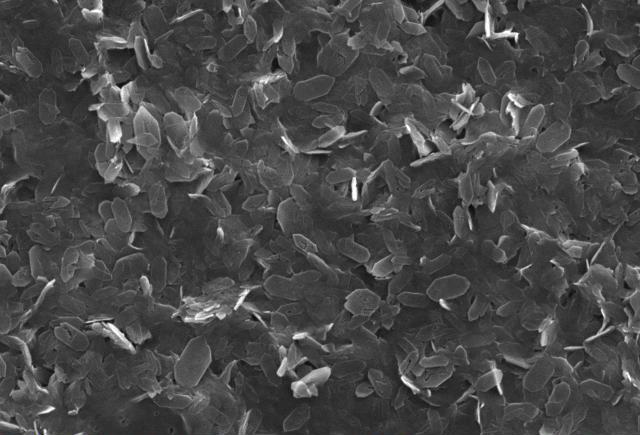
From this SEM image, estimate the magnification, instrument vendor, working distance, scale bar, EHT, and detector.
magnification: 3 K X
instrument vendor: JEOL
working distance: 23 mm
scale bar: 5000 nm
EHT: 20 kV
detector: SEI